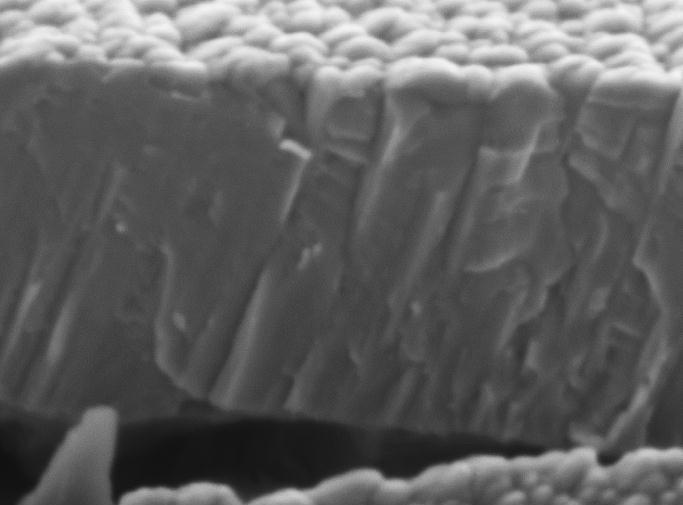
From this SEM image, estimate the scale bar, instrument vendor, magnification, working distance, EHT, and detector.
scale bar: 100 nm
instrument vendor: JEOL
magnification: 30 K X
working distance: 10.4 mm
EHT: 5 kV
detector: SEI